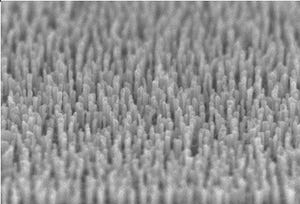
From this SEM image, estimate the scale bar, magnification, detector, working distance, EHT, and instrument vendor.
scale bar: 500 nm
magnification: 100 K X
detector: SE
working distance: -0.6 mm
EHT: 5 kV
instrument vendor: Hitachi